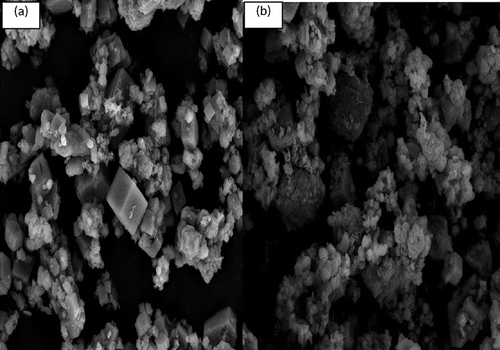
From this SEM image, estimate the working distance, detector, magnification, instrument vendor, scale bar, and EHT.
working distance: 10.4 mm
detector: LFD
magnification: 12 K X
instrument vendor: FEI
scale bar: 10000 nm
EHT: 10 kV